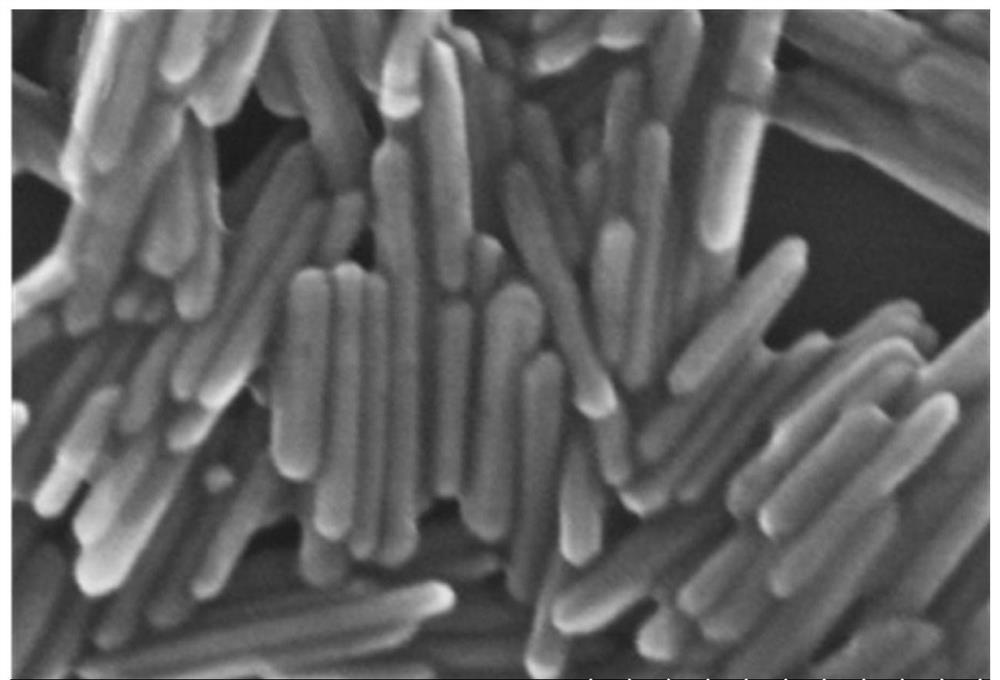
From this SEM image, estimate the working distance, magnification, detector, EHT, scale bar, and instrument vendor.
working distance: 8.9 mm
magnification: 250 K X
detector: SE(U)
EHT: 20 kV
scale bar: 200 nm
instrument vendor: Hitachi